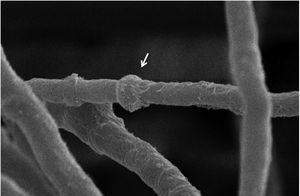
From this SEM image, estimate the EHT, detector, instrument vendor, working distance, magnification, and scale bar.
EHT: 15 kV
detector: SEI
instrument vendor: JEOL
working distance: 17 mm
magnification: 8 K X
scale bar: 2000 nm